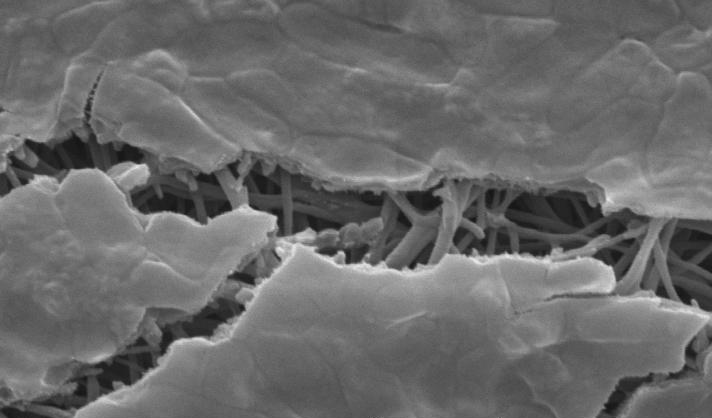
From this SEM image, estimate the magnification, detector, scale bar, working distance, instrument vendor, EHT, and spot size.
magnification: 8 K X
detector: SE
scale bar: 2000 nm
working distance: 7.8 mm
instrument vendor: FEI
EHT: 12 kV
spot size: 3